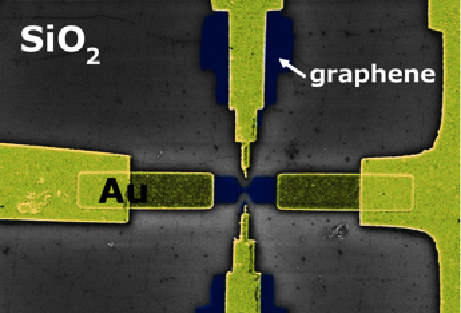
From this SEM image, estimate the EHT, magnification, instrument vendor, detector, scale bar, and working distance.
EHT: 5 kV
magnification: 8.23 K X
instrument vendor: Zeiss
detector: InLens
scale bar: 1000 nm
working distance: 5.1 mm